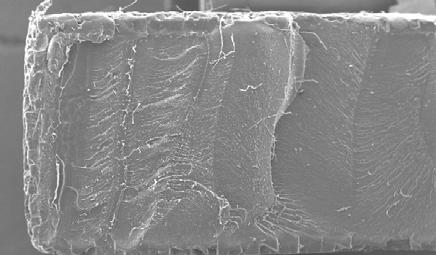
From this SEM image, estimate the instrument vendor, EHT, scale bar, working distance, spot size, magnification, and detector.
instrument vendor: FEI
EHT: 20 kV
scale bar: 1000000 nm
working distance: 10 mm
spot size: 5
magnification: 0.05 K X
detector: SE